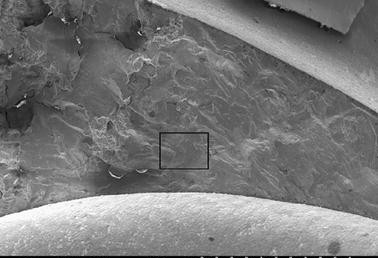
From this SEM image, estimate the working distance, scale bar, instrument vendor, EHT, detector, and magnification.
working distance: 38.6 mm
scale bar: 2000000 nm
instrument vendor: JEOL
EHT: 20 kV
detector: SE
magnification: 0.025 K X